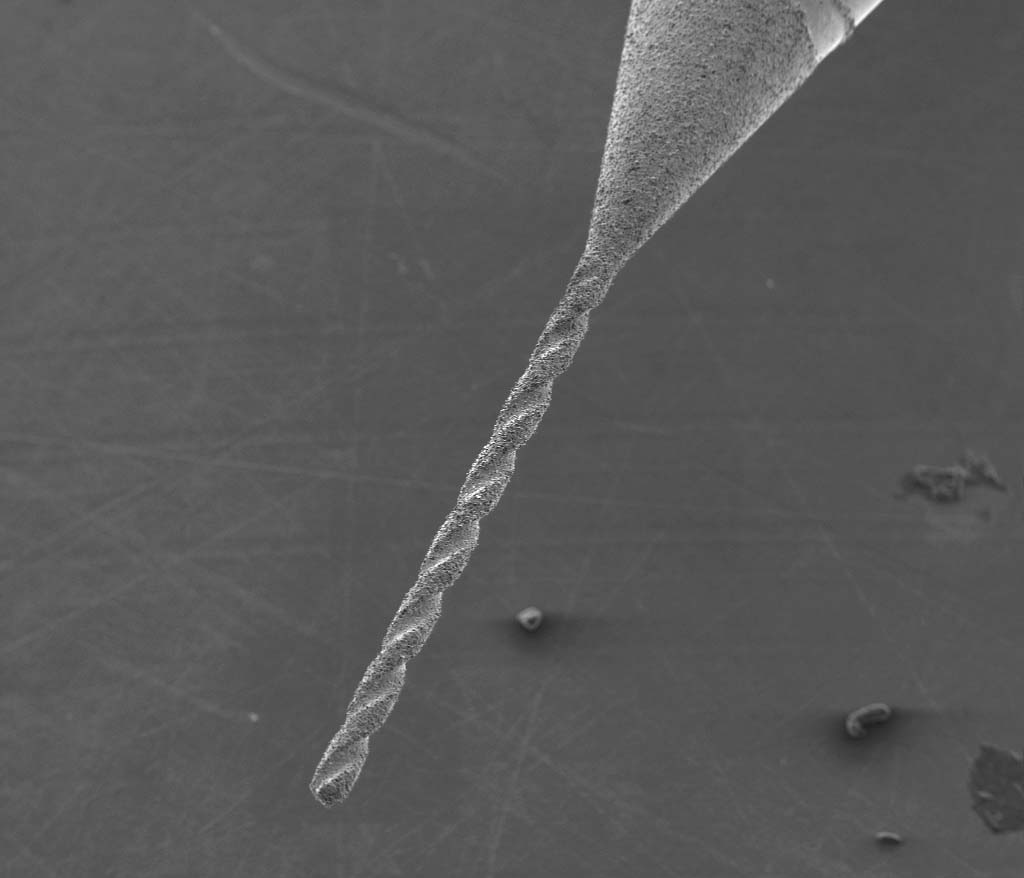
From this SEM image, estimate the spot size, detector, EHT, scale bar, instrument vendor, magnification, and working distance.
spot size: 5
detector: ETD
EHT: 15 kV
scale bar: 500000 nm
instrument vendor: FEI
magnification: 0.1 K X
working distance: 10.9 mm